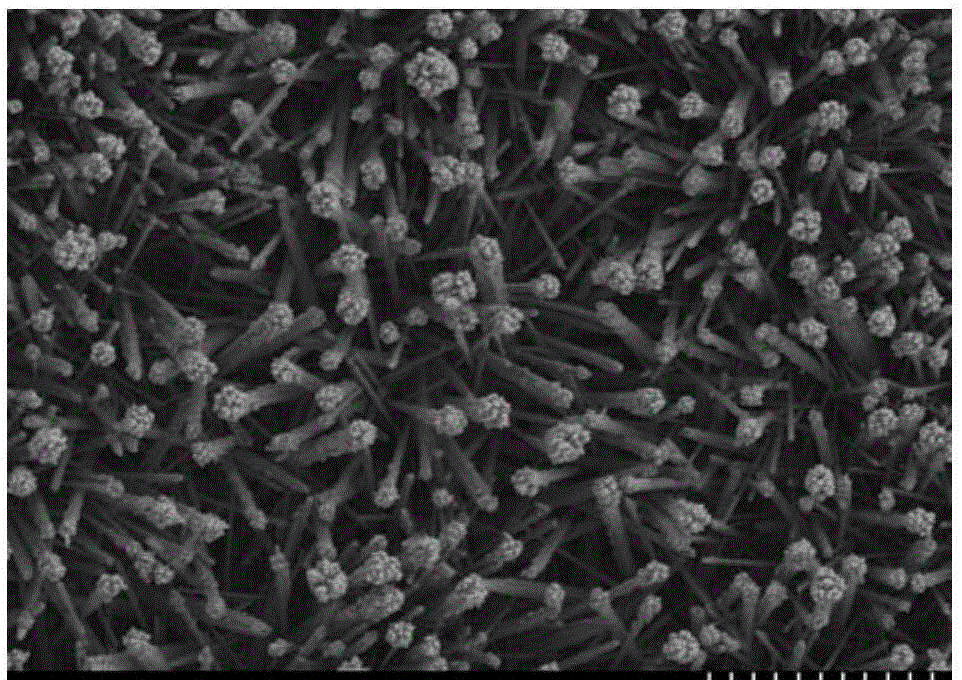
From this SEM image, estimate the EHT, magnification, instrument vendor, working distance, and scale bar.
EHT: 3 kV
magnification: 6 K X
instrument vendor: Hitachi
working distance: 17.3 mm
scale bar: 5000 nm